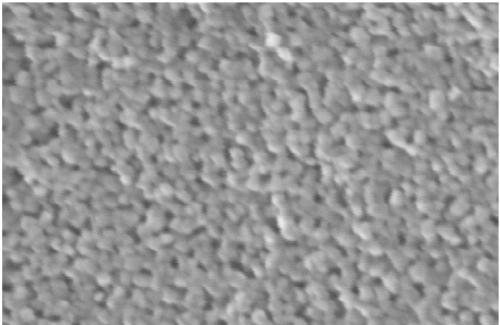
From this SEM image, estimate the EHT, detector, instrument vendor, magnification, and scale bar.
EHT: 20 kV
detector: InLens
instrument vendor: Zeiss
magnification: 250 K X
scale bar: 20 nm